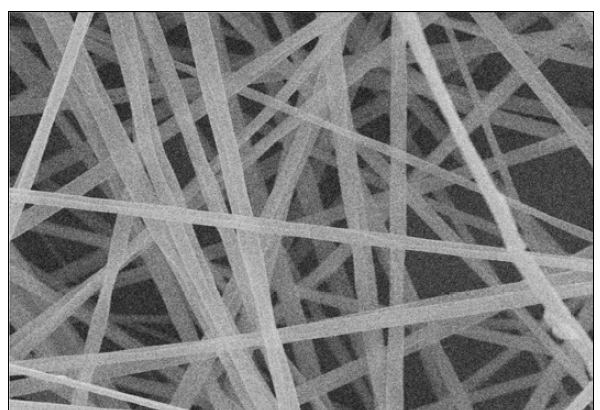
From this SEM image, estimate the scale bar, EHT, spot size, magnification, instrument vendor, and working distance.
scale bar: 15000 nm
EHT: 20 kV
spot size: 8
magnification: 2 K X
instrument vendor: other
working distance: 5 mm